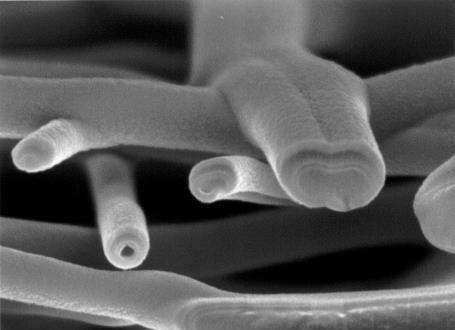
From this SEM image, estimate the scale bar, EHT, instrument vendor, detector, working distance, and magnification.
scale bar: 100 nm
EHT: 3 kV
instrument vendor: JEOL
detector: SEI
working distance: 2.9 mm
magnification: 70 K X